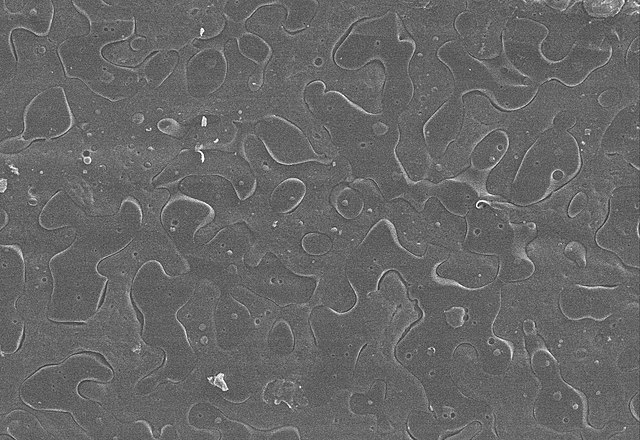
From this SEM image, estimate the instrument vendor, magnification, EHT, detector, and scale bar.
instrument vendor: other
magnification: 0.1 K X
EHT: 20 kV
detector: SE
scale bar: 300000 nm